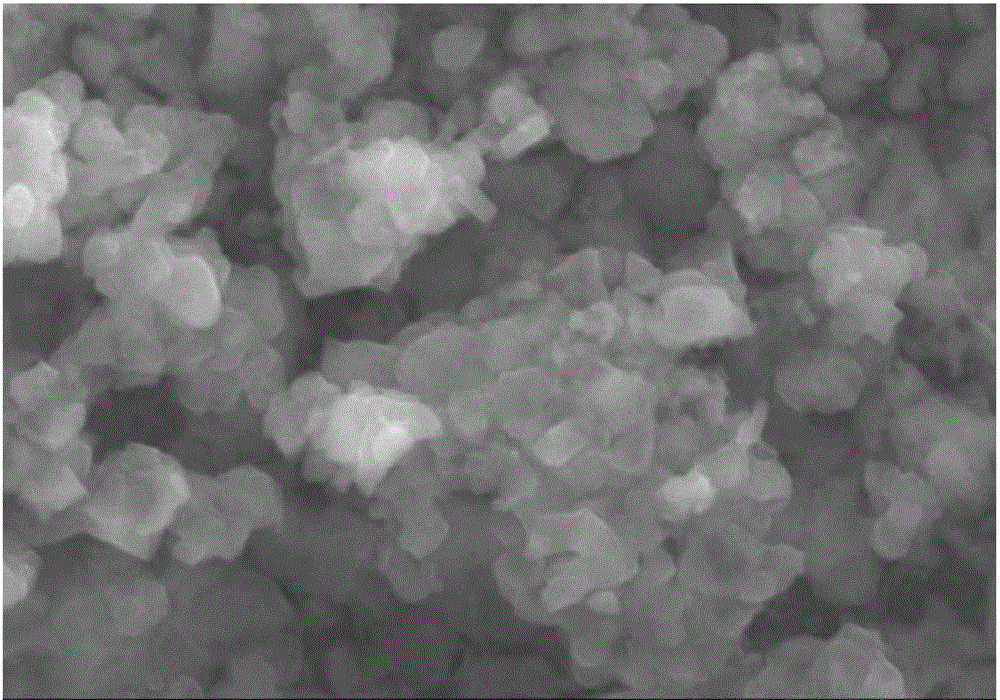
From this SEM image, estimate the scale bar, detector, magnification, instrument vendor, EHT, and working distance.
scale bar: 2000 nm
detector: SE(M)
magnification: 20 K X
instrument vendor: Hitachi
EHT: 20 kV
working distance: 19.3 mm